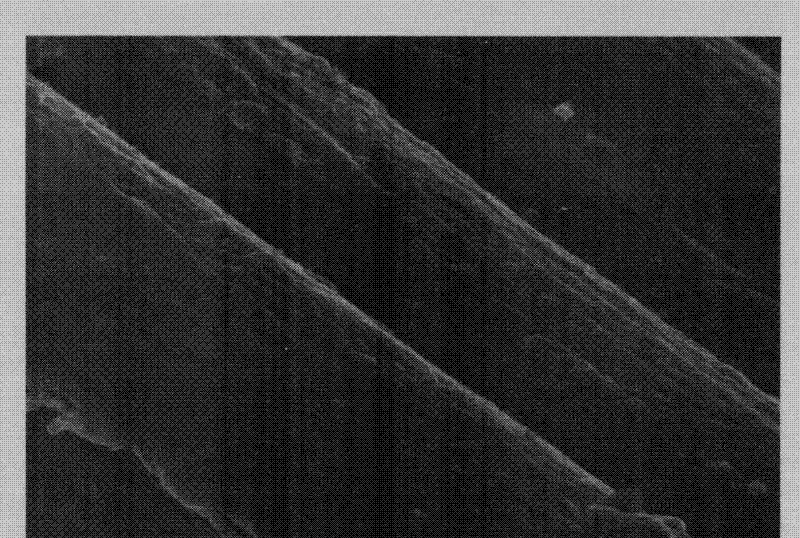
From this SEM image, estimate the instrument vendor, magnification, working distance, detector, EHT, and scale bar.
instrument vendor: Zeiss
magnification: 30 K X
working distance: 6.8 mm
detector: InLens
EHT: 2 kV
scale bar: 200 nm